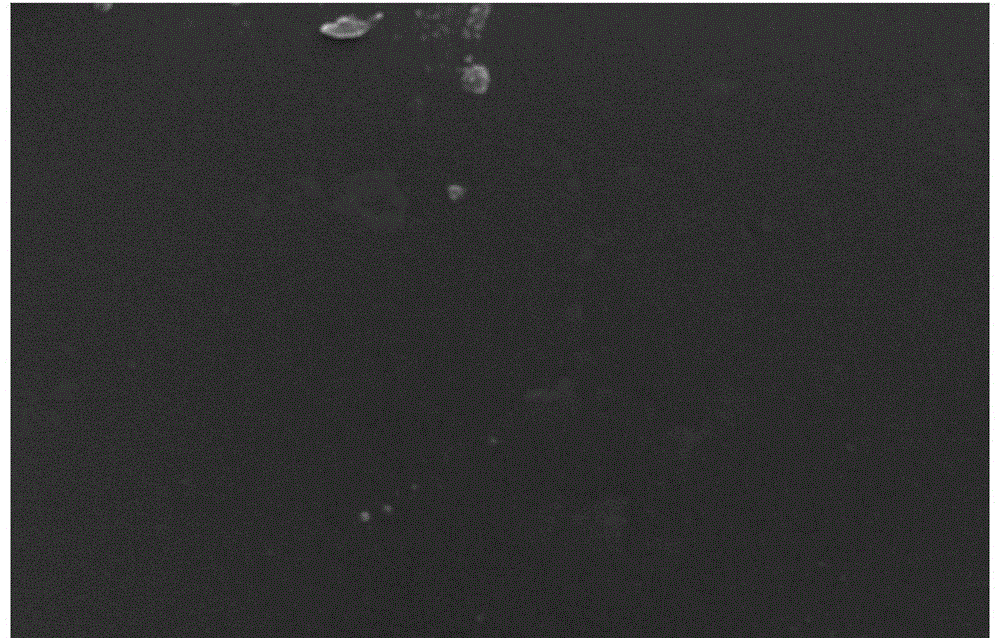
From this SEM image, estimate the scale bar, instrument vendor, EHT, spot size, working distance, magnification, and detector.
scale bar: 10000 nm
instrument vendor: FEI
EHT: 25 kV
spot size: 4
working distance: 7.1 mm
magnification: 5 K X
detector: SE